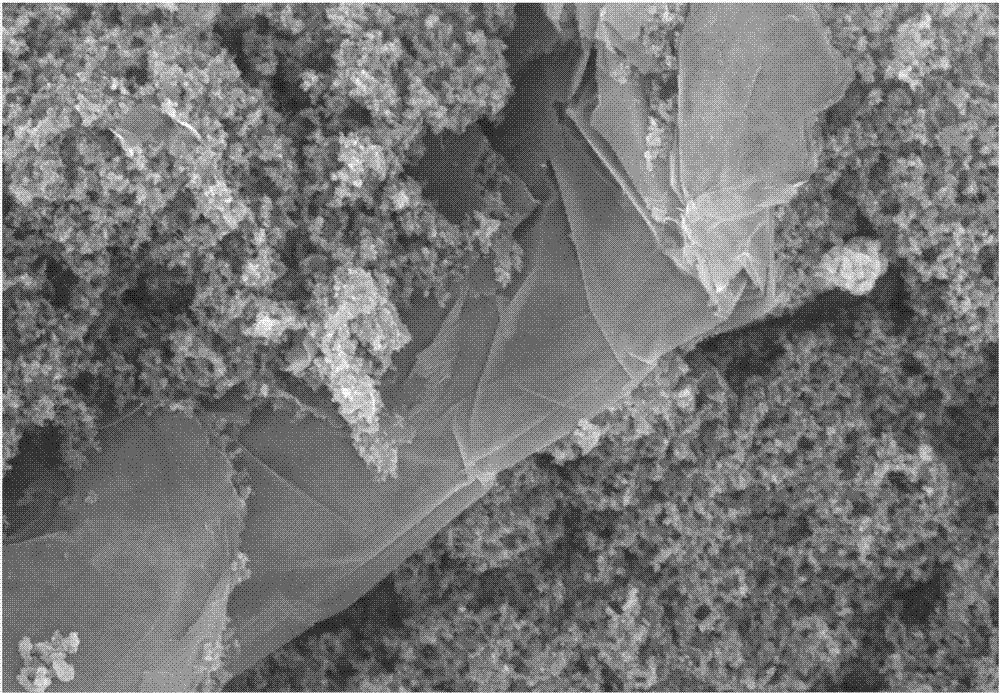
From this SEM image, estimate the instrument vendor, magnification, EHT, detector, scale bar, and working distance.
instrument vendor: Zeiss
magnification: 20 K X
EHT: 10 kV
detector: SE2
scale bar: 1000 nm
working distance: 6.5 mm